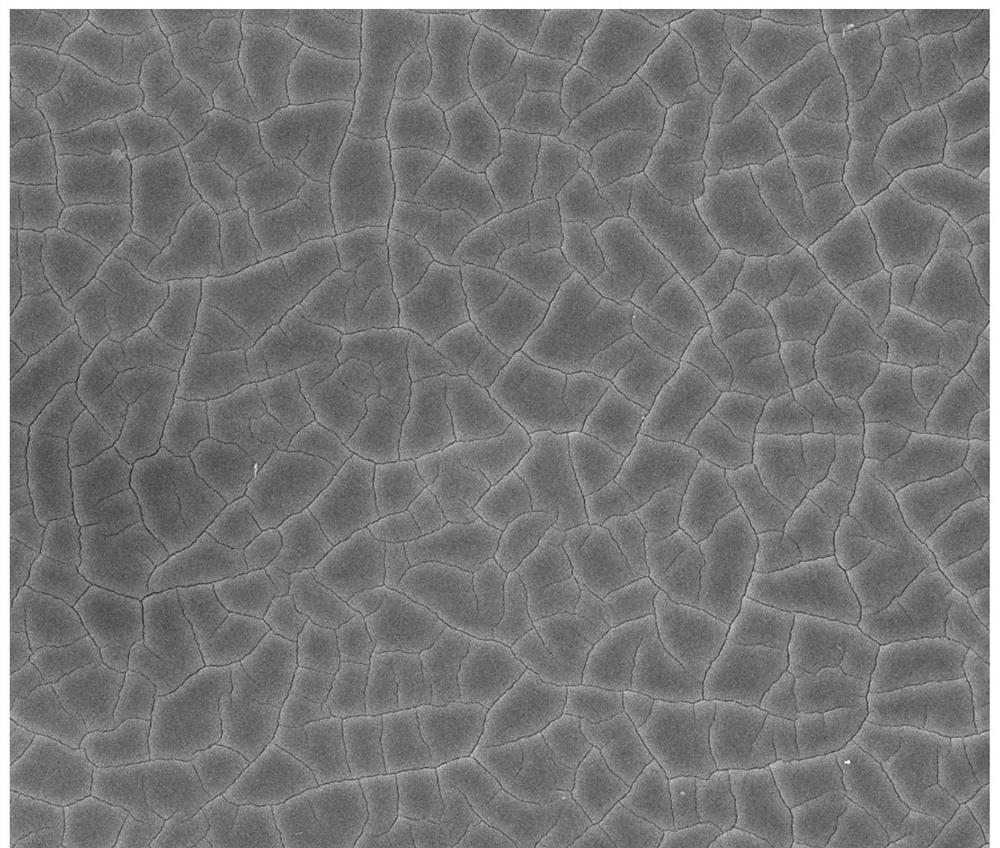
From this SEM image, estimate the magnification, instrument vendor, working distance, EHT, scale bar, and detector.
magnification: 5 K X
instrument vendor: FEI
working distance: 6.3 mm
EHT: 10 kV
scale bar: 10000 nm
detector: ETD